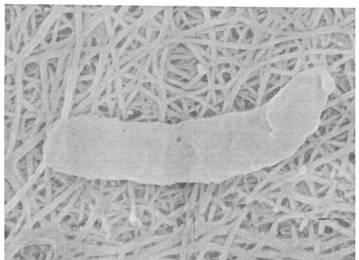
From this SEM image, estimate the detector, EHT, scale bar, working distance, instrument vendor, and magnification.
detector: SEI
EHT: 15 kV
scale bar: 100 nm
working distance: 16 mm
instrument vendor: JEOL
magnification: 30 K X